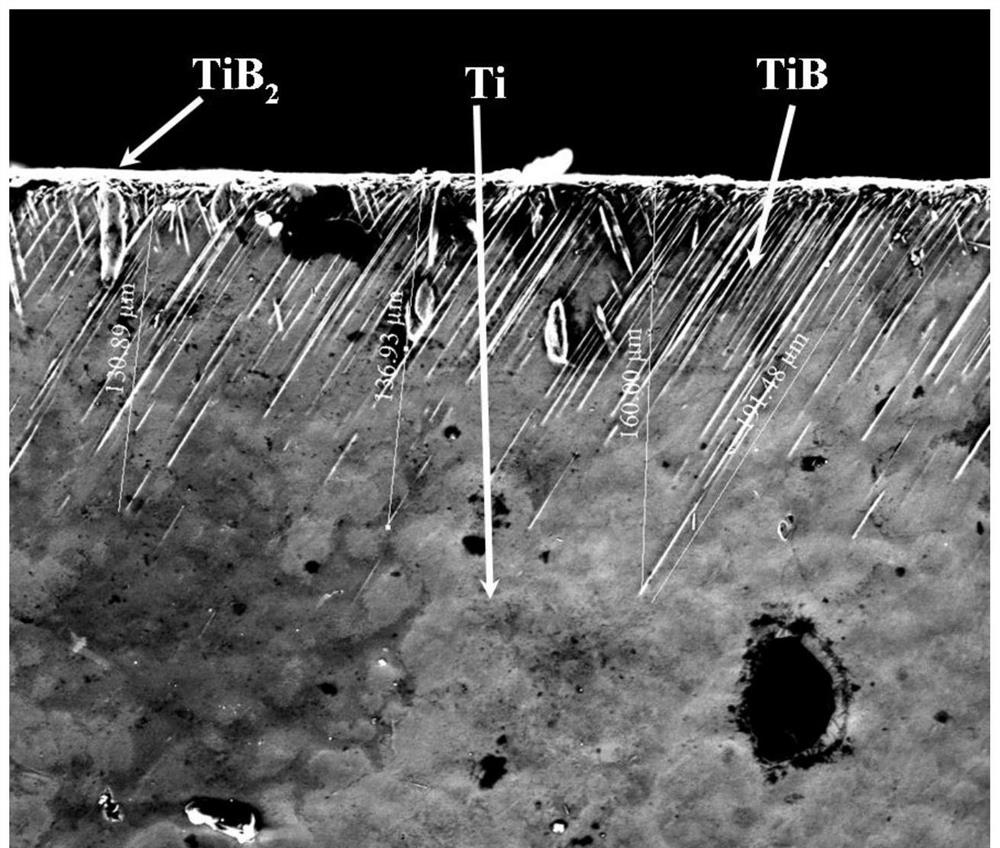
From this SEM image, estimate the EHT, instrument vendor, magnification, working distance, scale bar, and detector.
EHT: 20 kV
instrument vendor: FEI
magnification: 0.8 K X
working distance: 10.6 mm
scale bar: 100000 nm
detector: ETD